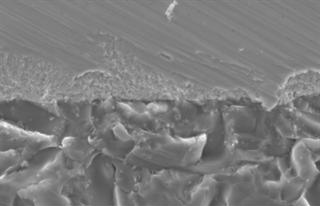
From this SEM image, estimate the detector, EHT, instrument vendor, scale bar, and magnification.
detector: SEI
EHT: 21 kV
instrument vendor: JEOL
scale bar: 10000 nm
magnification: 1.2 K X